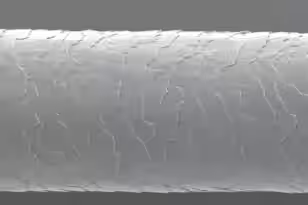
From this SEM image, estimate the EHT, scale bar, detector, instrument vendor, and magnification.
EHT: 5 kV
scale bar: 10000 nm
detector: SEI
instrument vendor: JEOL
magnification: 1.2 K X